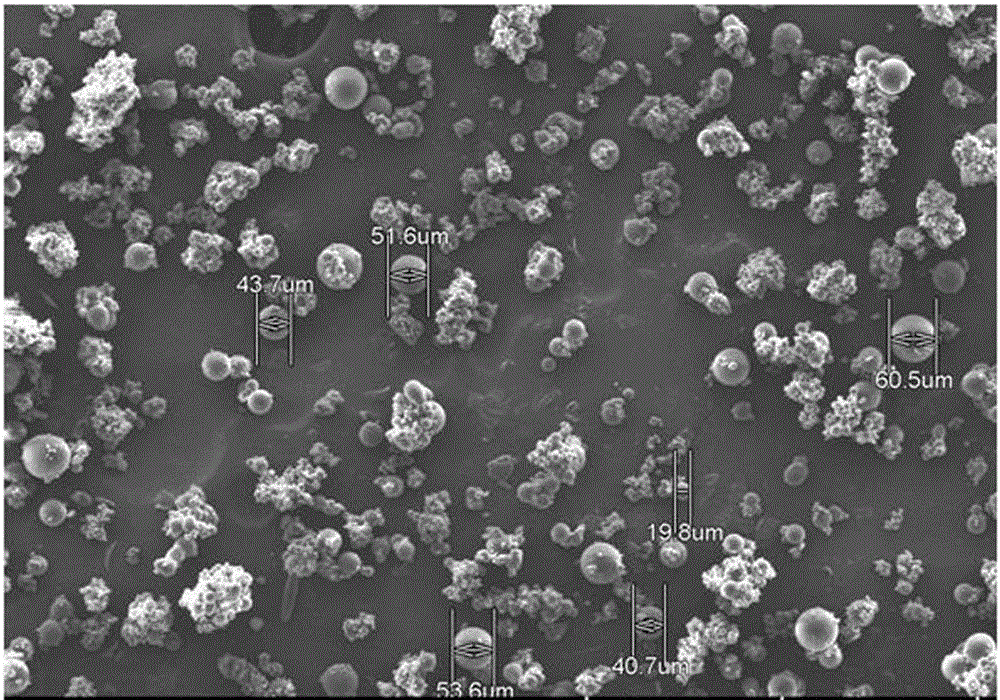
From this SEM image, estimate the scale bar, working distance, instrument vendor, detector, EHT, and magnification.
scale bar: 500000 nm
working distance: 7 mm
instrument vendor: Hitachi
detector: LM(L)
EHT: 15 kV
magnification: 0.1 K X